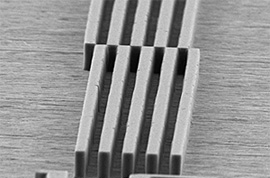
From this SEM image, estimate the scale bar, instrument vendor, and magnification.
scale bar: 20000 nm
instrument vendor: JEOL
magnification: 0.6 K X